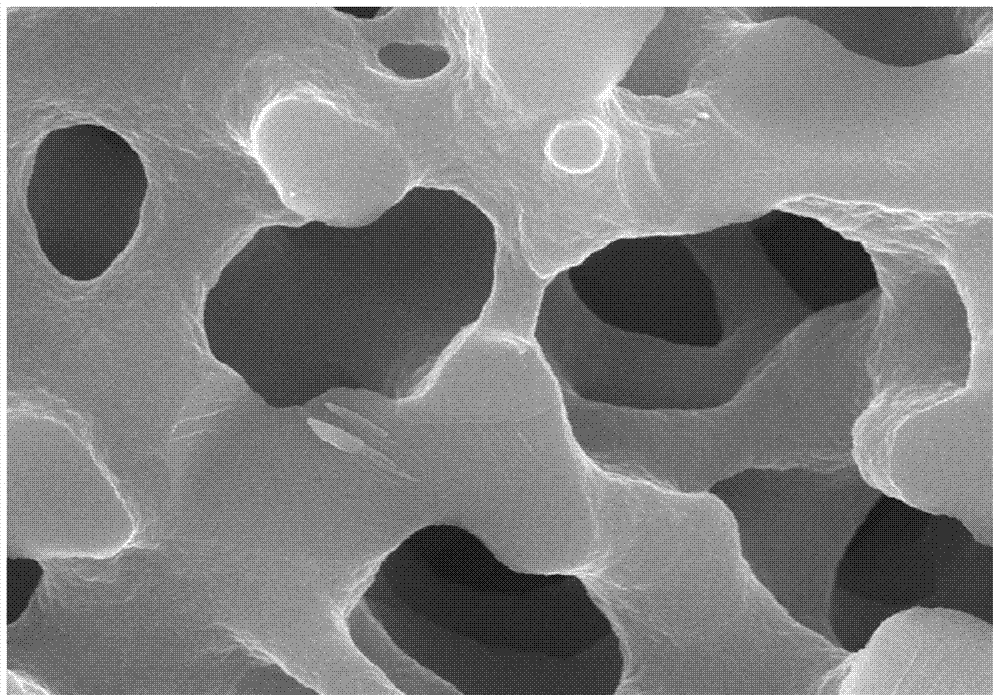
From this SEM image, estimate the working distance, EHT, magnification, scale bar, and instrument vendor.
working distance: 9.6 mm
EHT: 20 kV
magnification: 10 K X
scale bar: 5000 nm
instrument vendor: Hitachi